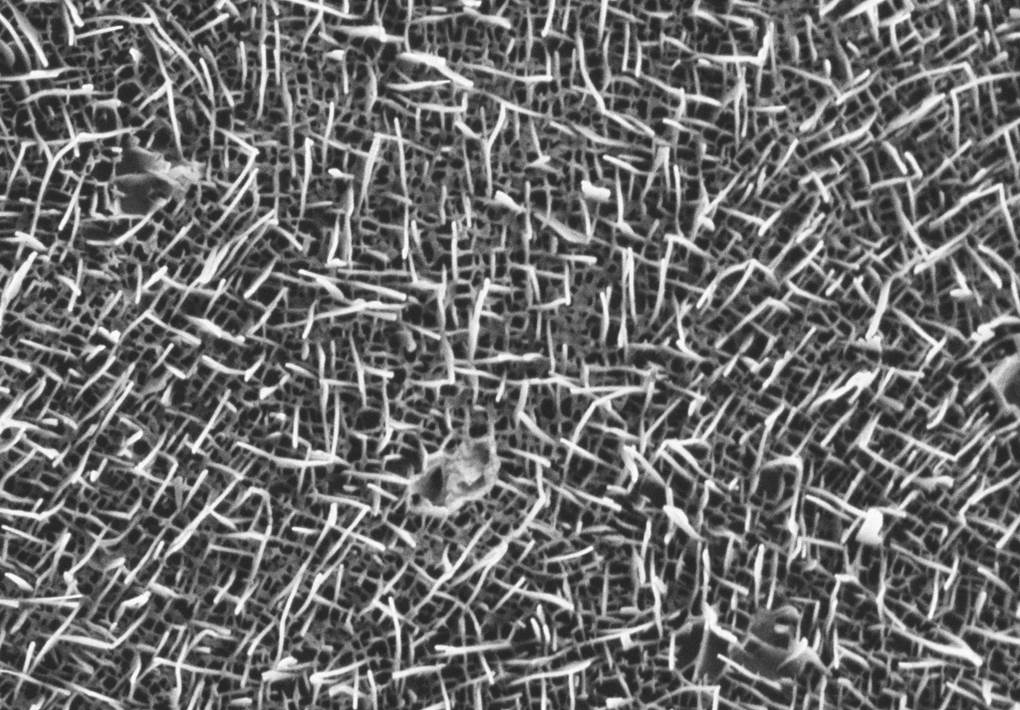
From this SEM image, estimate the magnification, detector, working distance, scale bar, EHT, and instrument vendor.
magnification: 20 K X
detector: SE2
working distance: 2.4 mm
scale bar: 1000 nm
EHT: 0.75 kV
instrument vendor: Zeiss